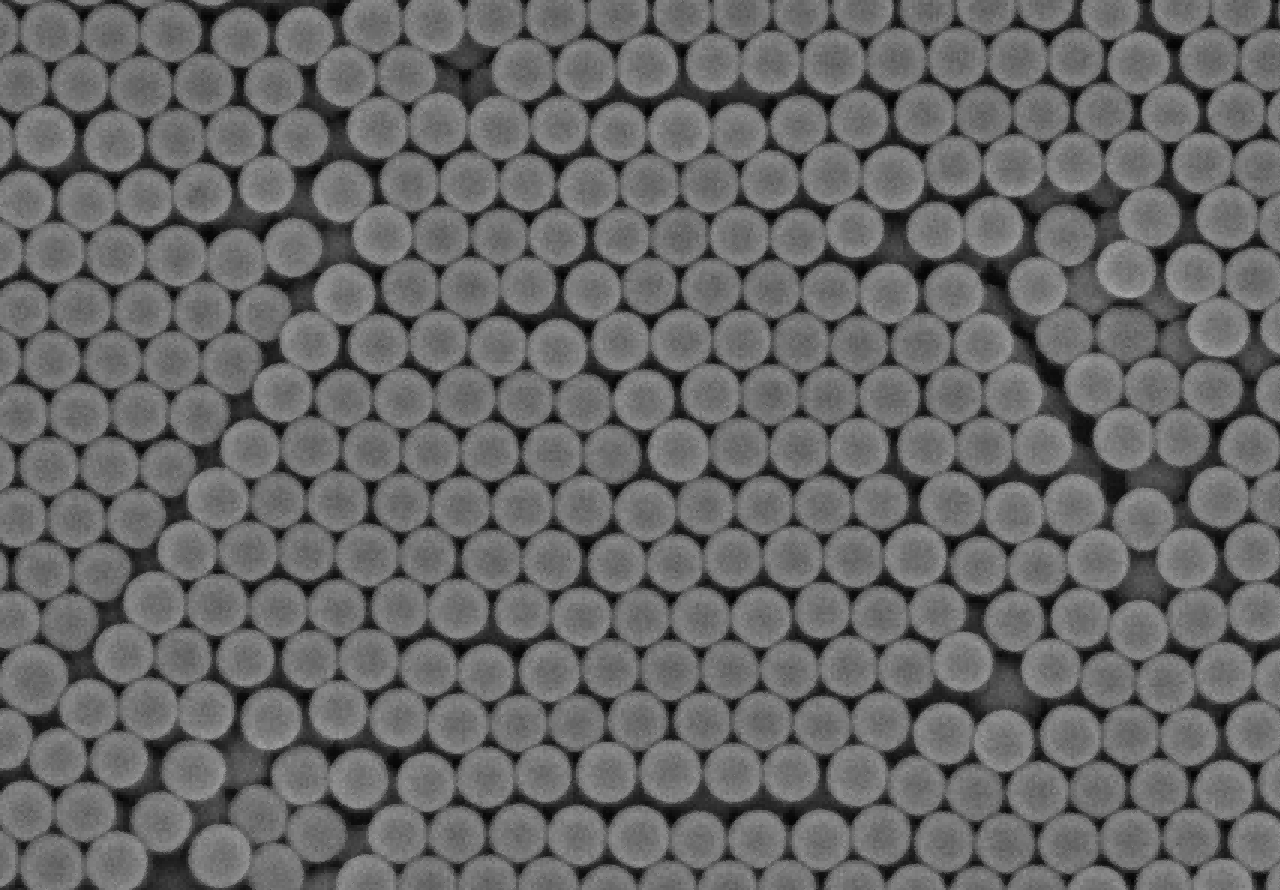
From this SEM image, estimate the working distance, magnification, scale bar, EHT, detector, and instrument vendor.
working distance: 3.7 mm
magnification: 30 K X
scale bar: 1000 nm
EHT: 3 kV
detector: SE(M)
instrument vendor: Hitachi